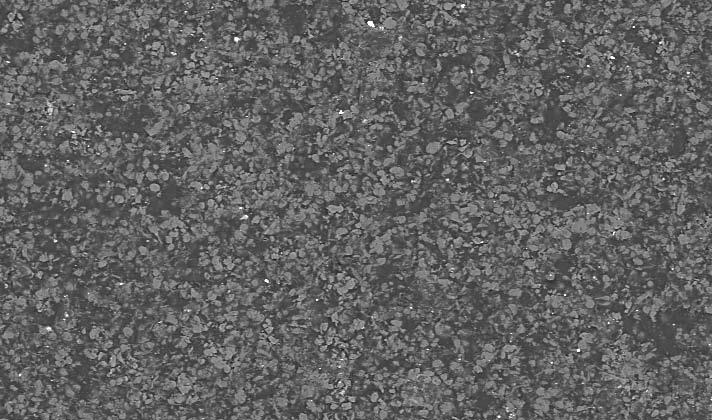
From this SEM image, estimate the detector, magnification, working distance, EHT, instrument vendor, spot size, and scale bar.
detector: BSE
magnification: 0.1 K X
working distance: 10.6 mm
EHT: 20 kV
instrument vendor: FEI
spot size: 4.7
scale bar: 200000 nm